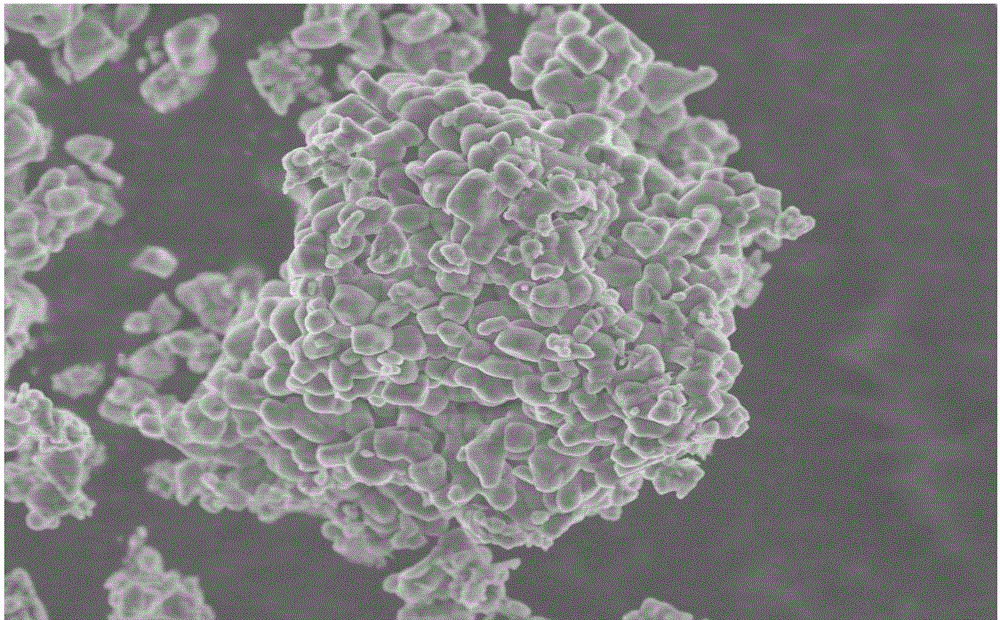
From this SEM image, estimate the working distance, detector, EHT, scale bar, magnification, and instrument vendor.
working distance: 8.2 mm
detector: SE(M)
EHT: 5 kV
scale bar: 10000 nm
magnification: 3 K X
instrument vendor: Hitachi